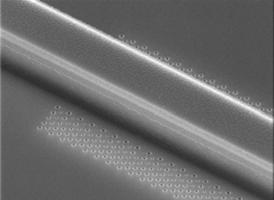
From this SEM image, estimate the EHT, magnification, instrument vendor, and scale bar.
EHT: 20 kV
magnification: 9 K X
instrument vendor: Hitachi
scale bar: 3330 nm